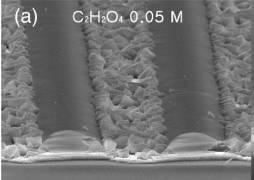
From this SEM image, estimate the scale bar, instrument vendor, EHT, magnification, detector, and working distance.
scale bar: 1000 nm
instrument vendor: JEOL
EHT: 15 kV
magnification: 3 K X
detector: SEI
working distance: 9.8 mm